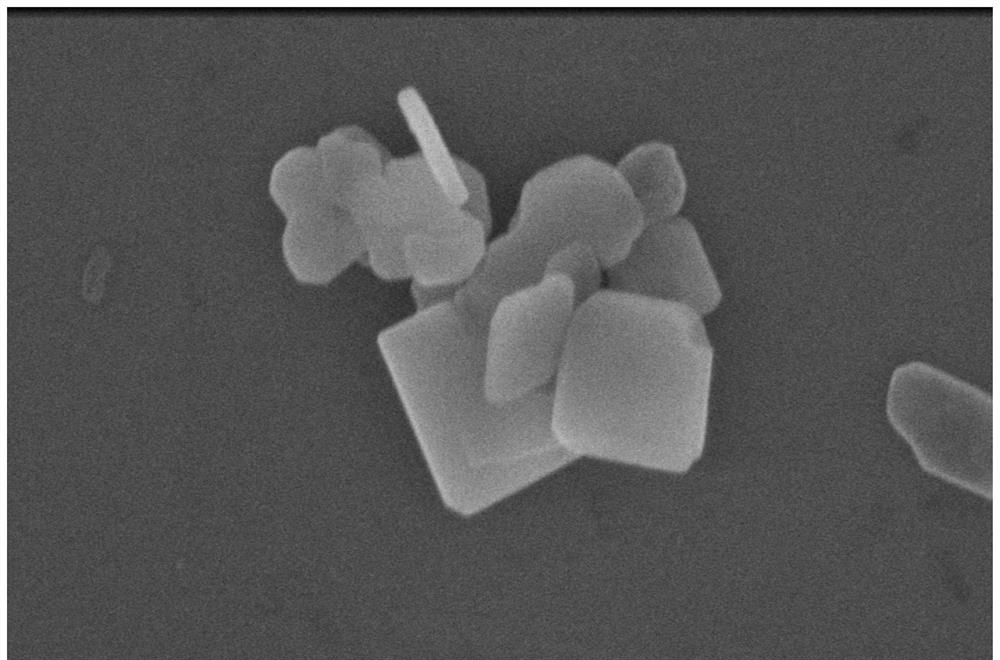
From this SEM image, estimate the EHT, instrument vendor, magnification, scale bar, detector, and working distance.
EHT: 5 kV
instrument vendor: FEI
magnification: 250 K X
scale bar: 500 nm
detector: TLD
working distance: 4.6 mm